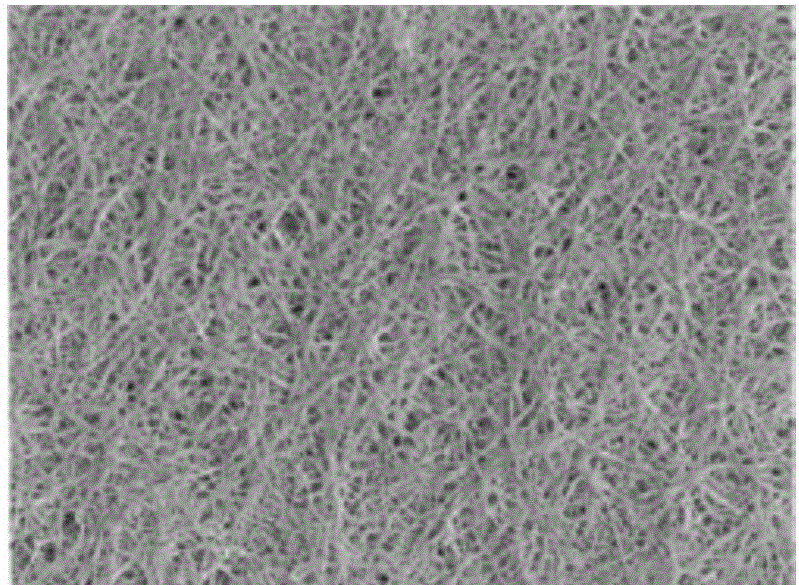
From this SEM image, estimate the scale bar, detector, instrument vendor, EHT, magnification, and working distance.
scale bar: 10000 nm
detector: SEI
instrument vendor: JEOL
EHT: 15 kV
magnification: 2 K X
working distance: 19 mm